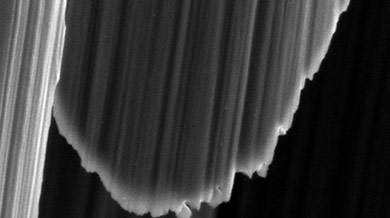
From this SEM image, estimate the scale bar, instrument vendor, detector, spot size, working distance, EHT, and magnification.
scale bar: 1000 nm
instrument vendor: FEI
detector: SE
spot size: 3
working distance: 5.6 mm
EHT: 3 kV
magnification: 19.362 K X